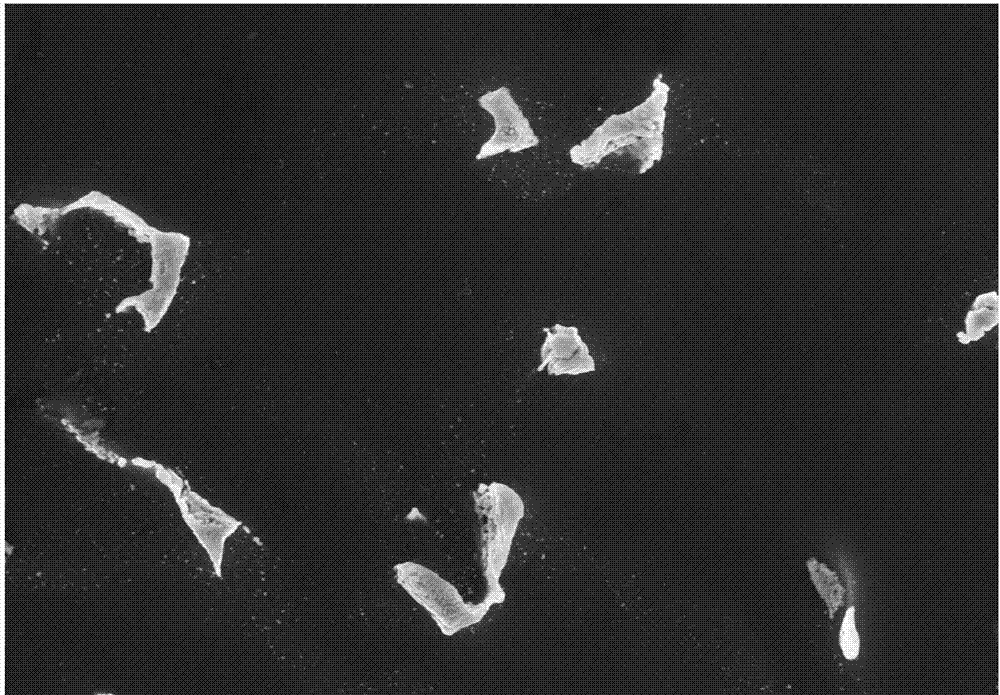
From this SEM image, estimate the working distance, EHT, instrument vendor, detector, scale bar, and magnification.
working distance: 7.4 mm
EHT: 15 kV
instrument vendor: Hitachi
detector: SE(M)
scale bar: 20000 nm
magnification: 2 K X